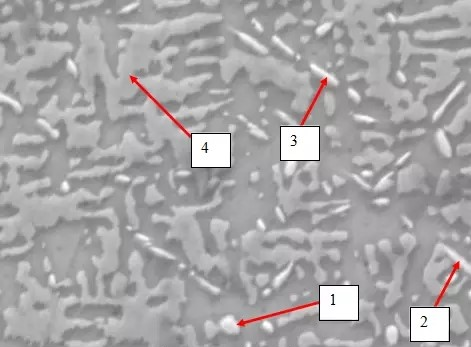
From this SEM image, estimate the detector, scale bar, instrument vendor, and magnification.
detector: SEI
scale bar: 5000 nm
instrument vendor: JEOL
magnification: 4 K X